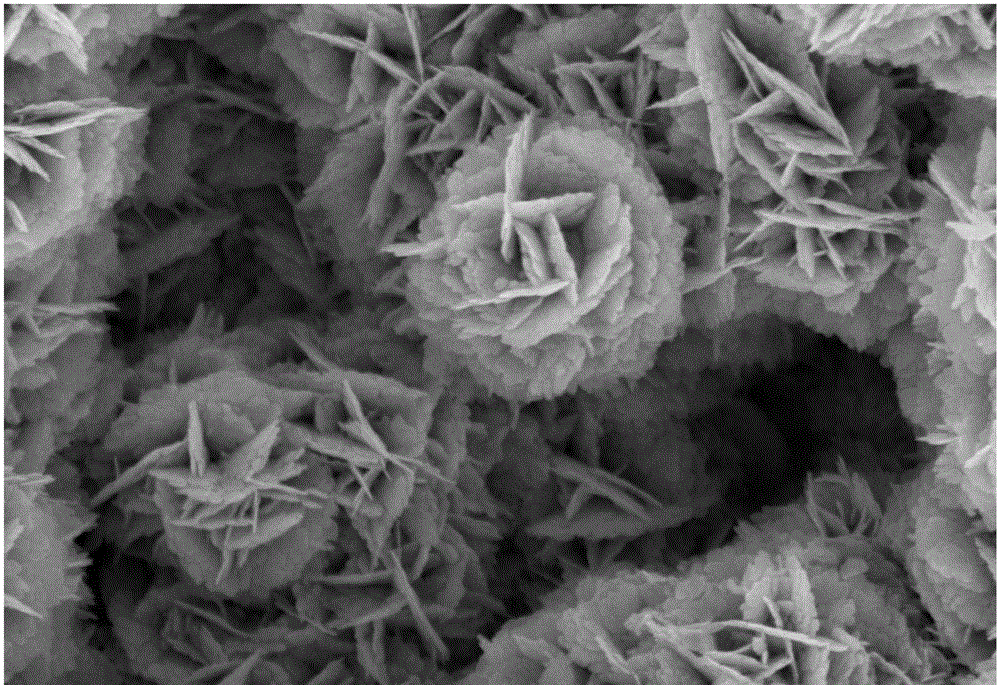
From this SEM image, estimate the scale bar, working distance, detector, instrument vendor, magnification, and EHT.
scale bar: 200 nm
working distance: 6 mm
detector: InLens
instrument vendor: Zeiss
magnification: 50 K X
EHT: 5 kV